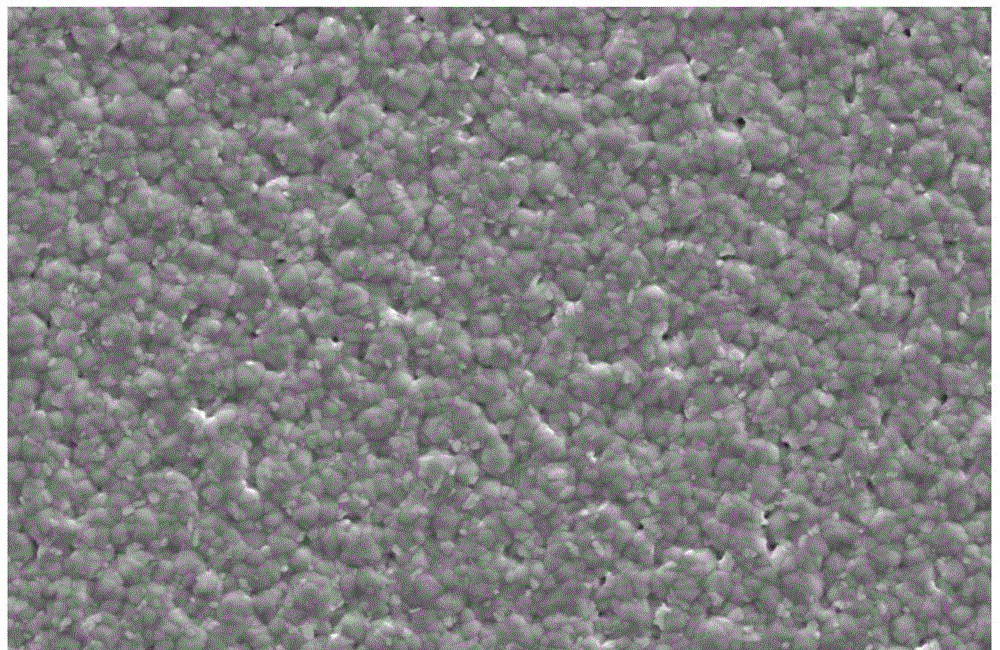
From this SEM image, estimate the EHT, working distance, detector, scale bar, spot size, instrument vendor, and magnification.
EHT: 5 kV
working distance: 5.1 mm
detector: SE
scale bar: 2000 nm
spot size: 4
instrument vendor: FEI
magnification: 20 K X